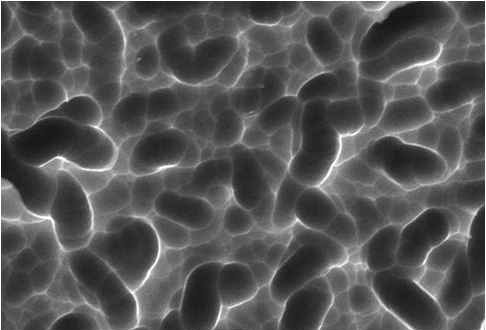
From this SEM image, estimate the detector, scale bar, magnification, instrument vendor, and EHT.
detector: SEI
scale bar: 5000 nm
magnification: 3 K X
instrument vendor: JEOL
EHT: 20 kV